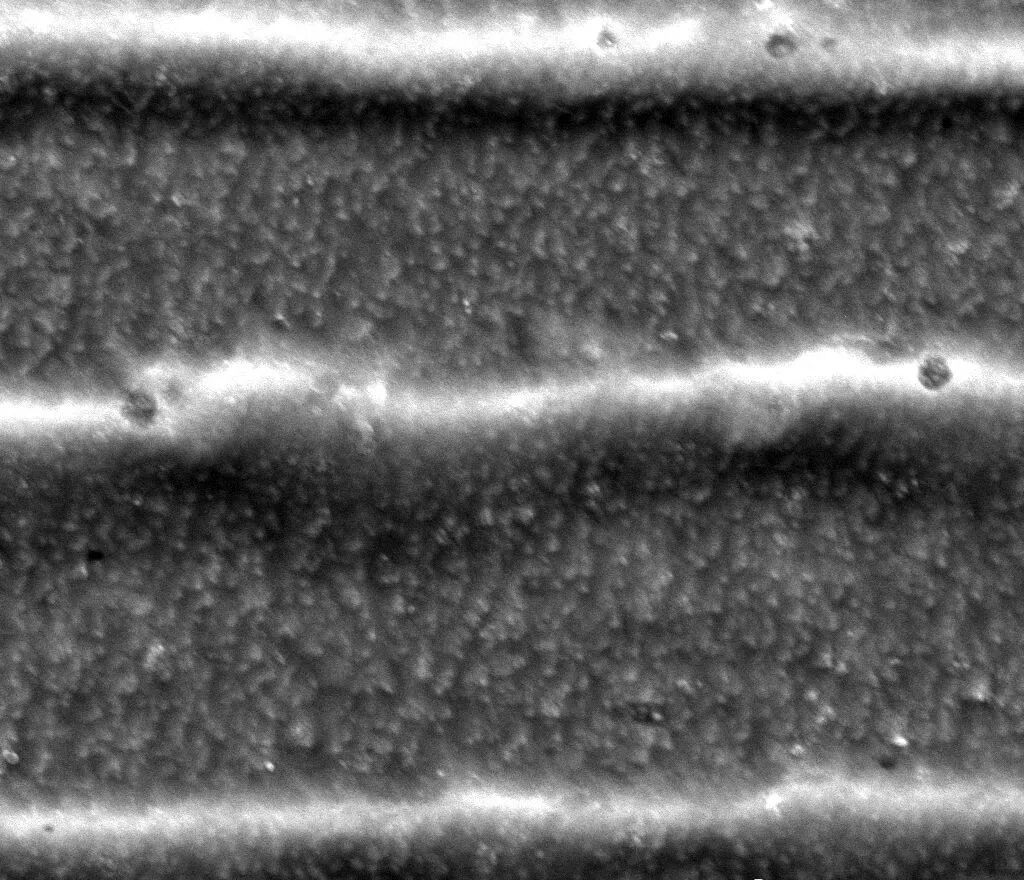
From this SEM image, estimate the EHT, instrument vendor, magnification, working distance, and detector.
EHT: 20 kV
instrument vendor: FEI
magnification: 10 K X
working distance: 8 mm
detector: ETD